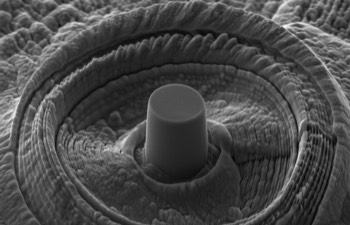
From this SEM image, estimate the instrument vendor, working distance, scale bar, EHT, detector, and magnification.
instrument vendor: Zeiss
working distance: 6.5 mm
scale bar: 1000 nm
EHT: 5 kV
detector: SE2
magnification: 10 K X